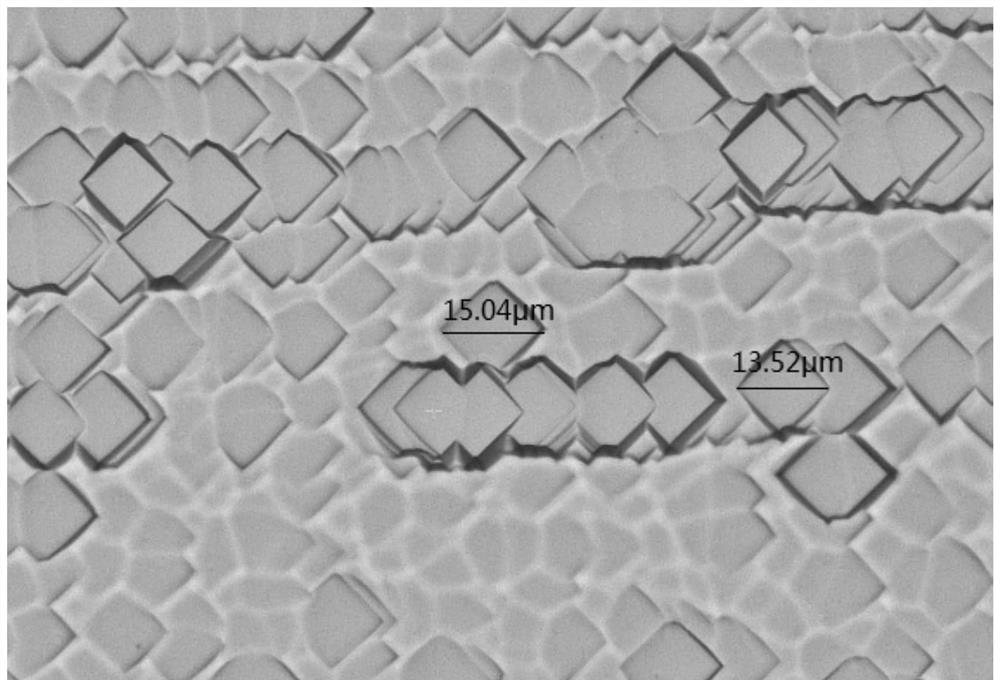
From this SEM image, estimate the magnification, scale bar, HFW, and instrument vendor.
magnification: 0.05 K X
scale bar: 15000 nm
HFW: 177 µm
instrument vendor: other